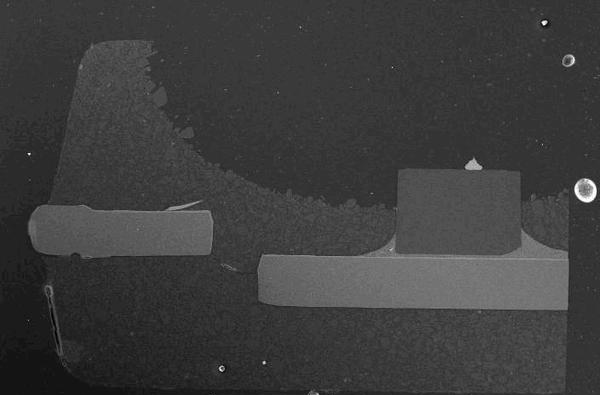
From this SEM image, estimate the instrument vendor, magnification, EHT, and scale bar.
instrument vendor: JEOL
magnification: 0.05 K X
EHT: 20 kV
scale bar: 500000 nm